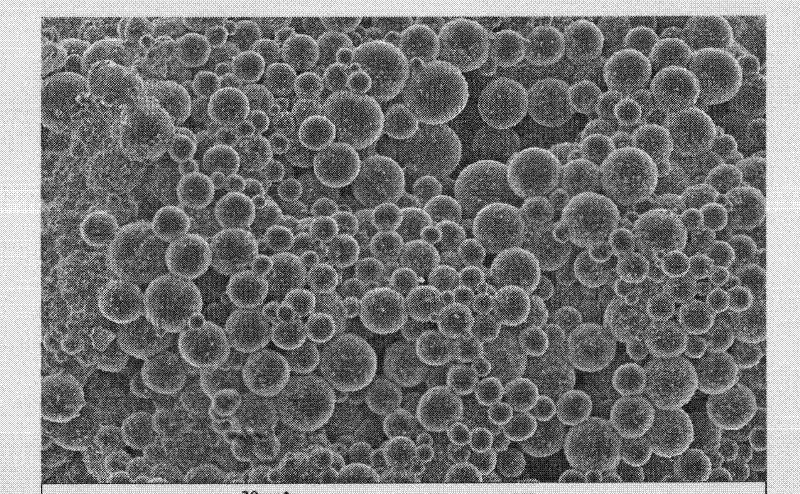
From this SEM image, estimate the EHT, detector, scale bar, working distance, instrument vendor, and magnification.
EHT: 10 kV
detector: InLens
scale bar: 30000 nm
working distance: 6 mm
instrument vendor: Zeiss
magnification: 0.8 K X